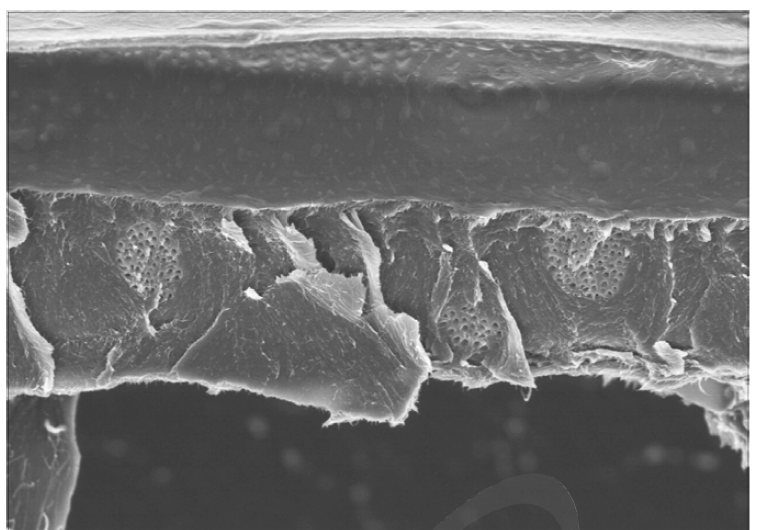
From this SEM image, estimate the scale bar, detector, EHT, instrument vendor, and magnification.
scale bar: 5000 nm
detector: SE(M)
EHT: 3 kV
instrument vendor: Hitachi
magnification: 10 K X